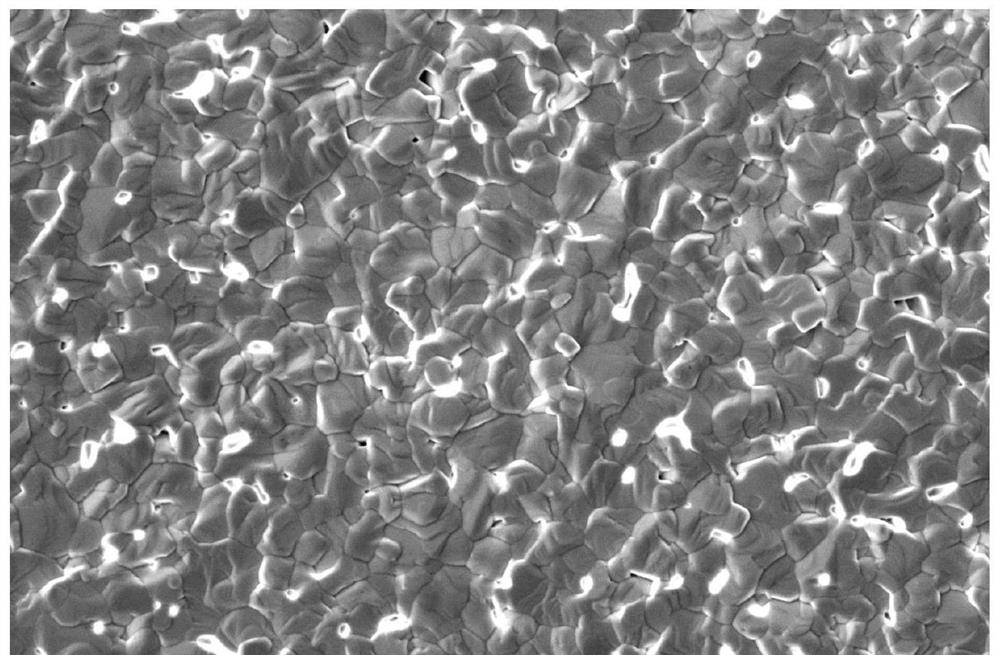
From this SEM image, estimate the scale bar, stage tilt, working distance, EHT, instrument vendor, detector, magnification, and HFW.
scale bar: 5000 nm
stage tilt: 0°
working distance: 7.7 mm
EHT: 2 kV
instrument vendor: Thermo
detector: ETD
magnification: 10 K X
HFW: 12.7 µm